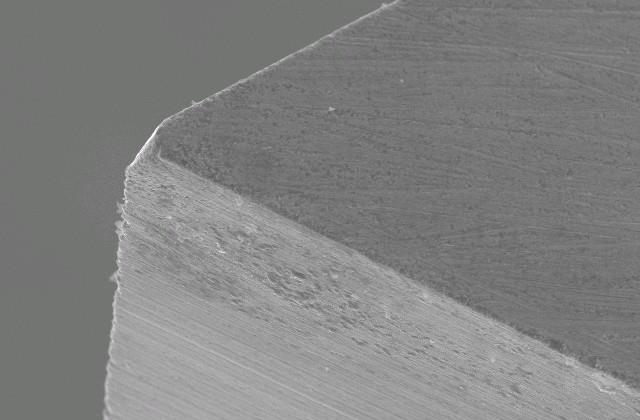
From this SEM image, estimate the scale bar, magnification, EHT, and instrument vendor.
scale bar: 50000 nm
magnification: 0.5 K X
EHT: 20 kV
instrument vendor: JEOL